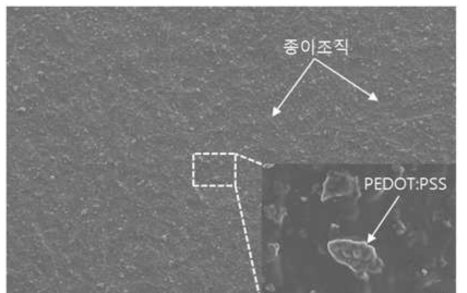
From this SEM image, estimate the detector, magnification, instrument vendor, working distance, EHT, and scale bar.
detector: SE(M)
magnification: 0.05 K X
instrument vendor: Hitachi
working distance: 11.6 mm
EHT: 7 kV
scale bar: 1000000 nm